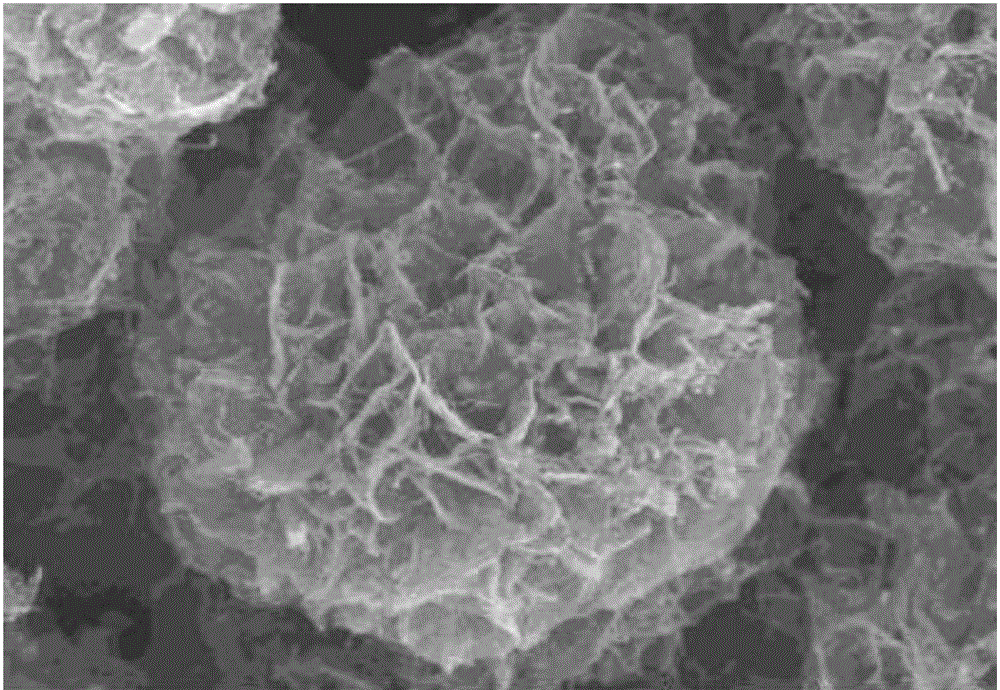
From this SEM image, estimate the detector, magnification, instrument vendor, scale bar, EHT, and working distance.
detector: SE(U)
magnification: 20 K X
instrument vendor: Hitachi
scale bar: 2000 nm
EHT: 10 kV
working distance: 8.2 mm